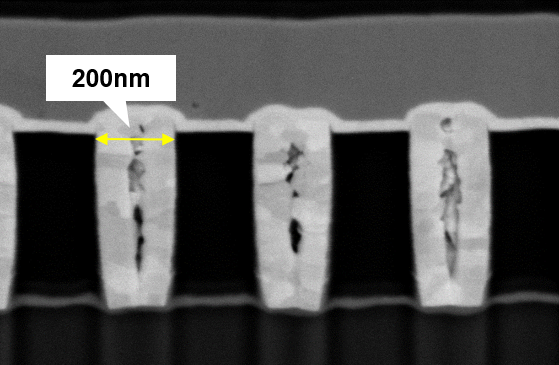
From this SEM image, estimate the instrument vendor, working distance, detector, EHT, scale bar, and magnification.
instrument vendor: FEI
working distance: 4 mm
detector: TLD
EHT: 2 kV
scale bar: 500 nm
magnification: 100 K X